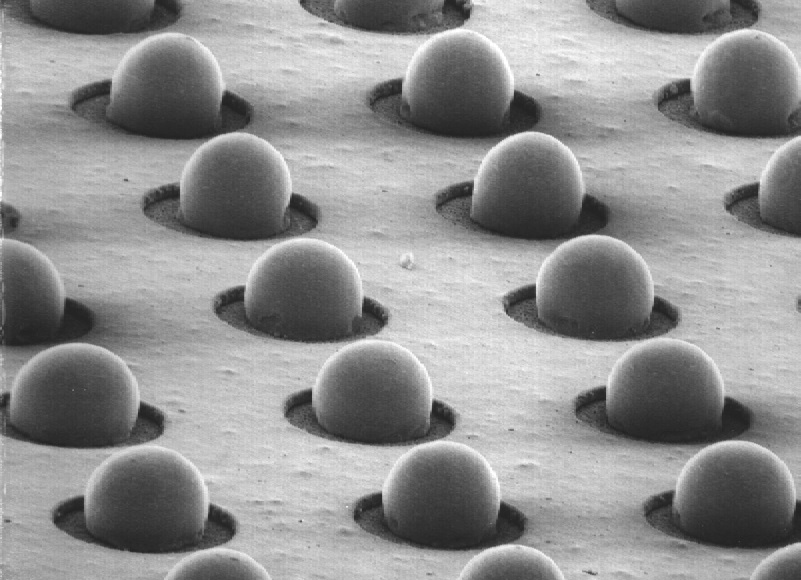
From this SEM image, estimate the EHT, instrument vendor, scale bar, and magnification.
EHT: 20 kV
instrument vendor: JEOL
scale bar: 132000 nm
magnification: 0.076 K X